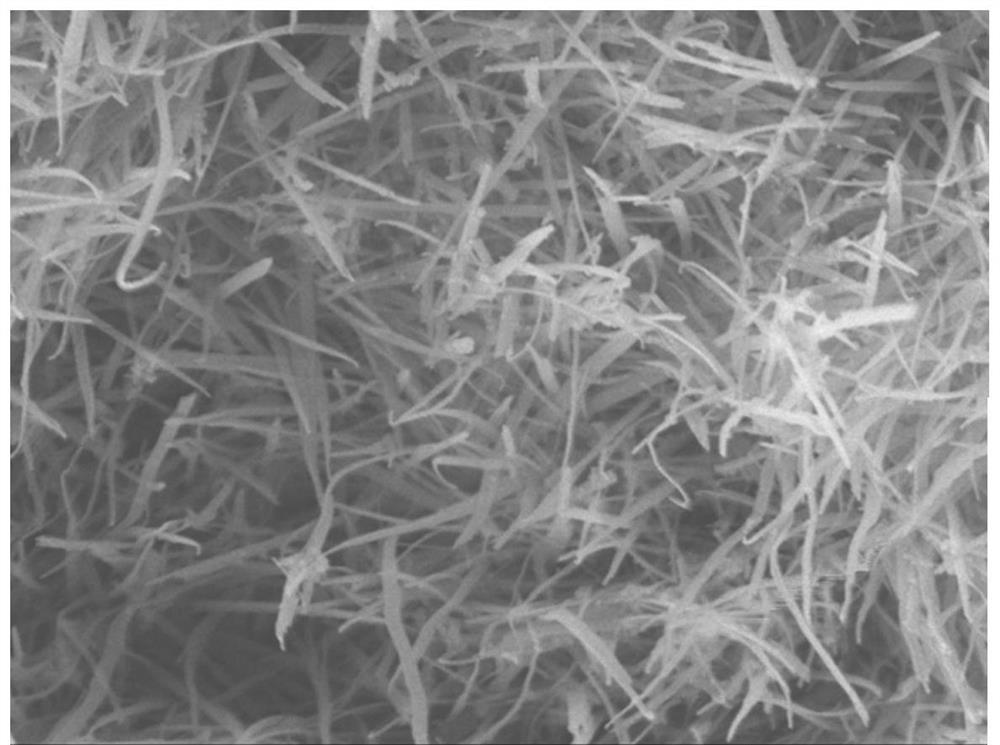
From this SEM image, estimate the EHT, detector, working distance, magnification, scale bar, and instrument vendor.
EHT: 5 kV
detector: LEI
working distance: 7.9 mm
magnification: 30 K X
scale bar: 100 nm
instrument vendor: JEOL